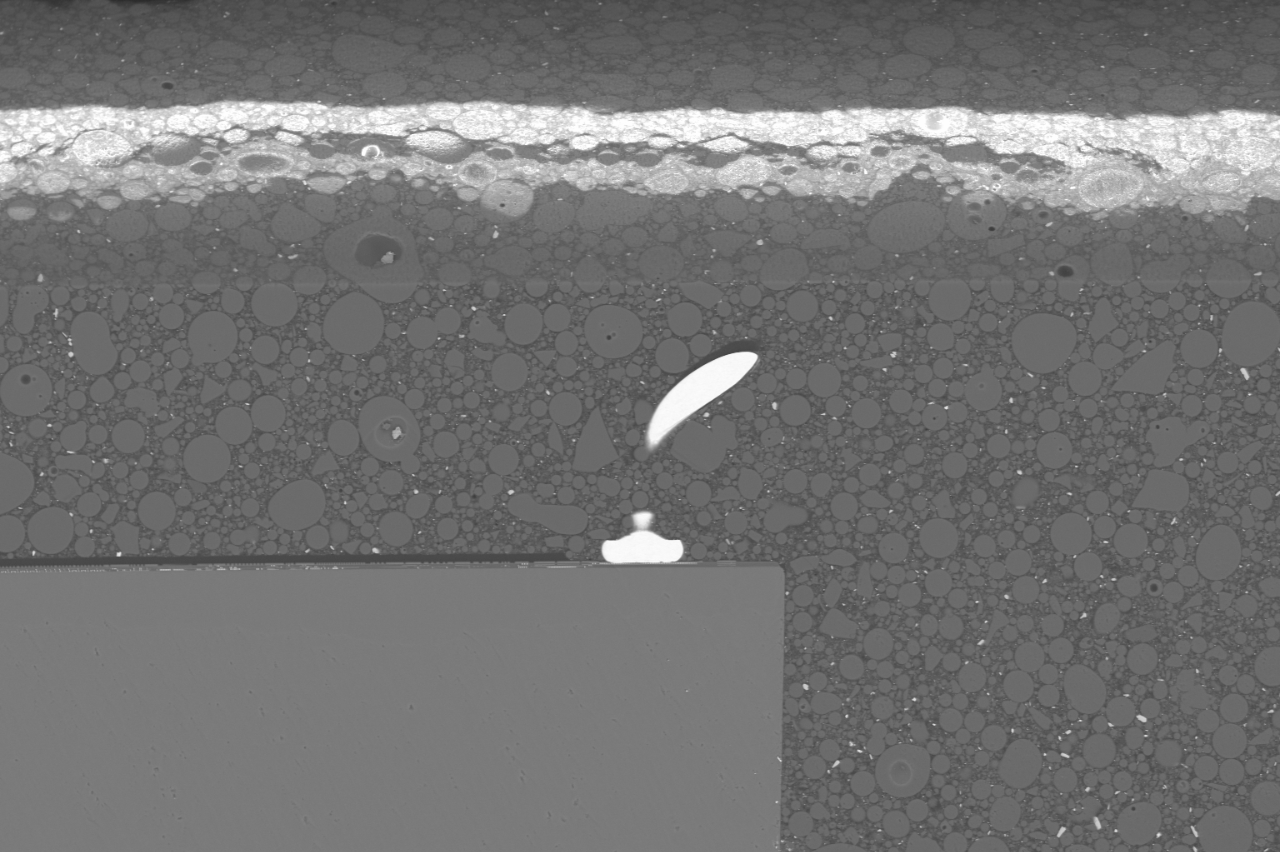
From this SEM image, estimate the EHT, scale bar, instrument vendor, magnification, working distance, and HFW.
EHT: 20 kV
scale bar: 500000 nm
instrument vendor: other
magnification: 0.4 K X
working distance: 5 mm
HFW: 1040 µm